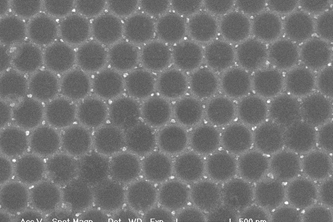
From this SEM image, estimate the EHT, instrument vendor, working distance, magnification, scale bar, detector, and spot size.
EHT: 30 kV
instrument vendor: FEI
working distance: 5 mm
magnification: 40 K X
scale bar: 500 nm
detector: TLD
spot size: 1